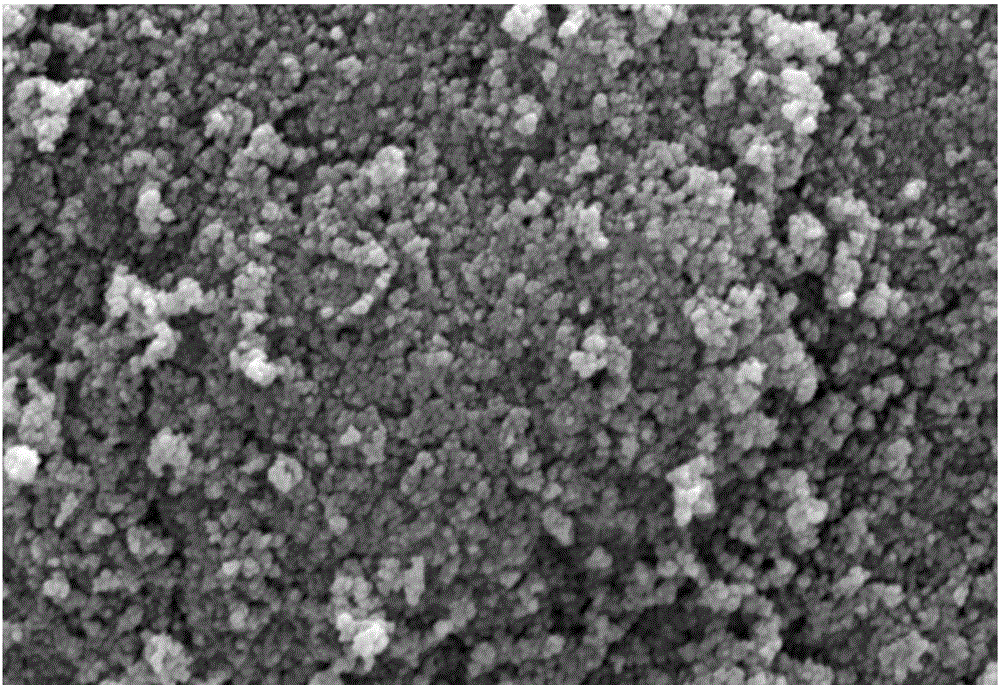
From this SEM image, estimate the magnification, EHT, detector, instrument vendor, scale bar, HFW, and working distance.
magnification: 80 K X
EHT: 10 kV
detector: ETD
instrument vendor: FEI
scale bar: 1000 nm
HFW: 5.18 µm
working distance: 9.3 mm